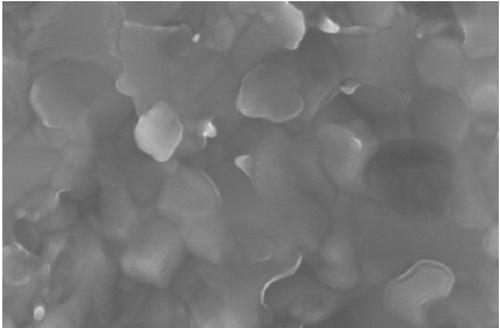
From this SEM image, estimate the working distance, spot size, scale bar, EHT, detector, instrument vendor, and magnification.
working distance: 6.1 mm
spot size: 3.5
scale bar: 500 nm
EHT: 15 kV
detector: TLD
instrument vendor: FEI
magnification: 300 K X